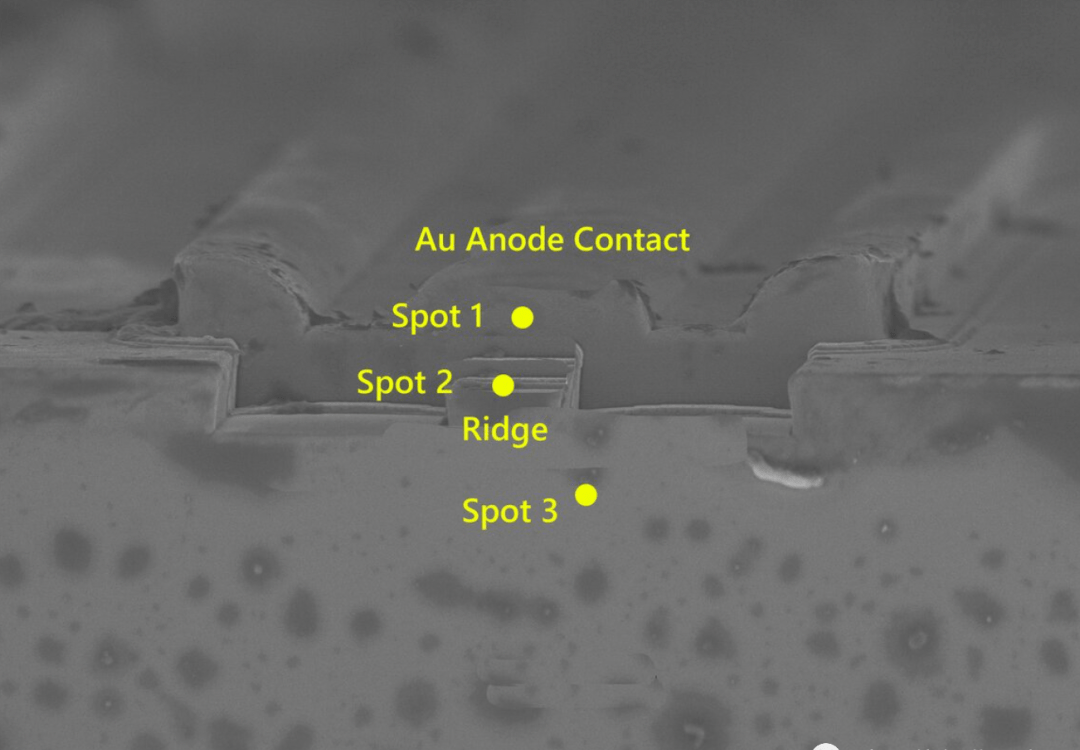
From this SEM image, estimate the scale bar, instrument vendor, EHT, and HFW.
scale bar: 2000 nm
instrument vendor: Zeiss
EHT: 5 kV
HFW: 36.47 µm